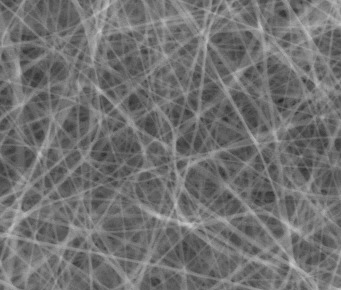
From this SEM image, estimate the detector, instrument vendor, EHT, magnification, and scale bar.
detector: LFD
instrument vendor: FEI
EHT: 20 kV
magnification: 10 K X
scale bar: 10000 nm